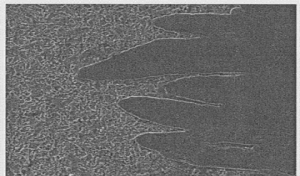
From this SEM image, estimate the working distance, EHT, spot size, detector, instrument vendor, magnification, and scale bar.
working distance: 9.3 mm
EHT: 30 kV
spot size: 2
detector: ETD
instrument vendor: FEI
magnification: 0.5 K X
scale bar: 100000 nm